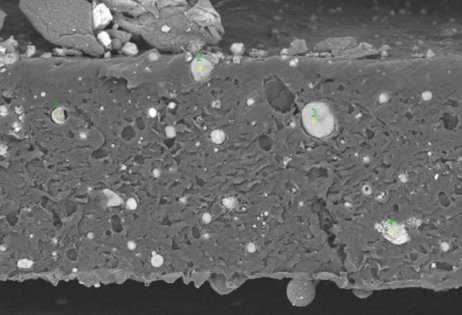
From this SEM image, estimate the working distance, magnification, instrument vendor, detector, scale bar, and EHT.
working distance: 8.4 mm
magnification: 2 K X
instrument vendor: other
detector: SE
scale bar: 30000 nm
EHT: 10 kV